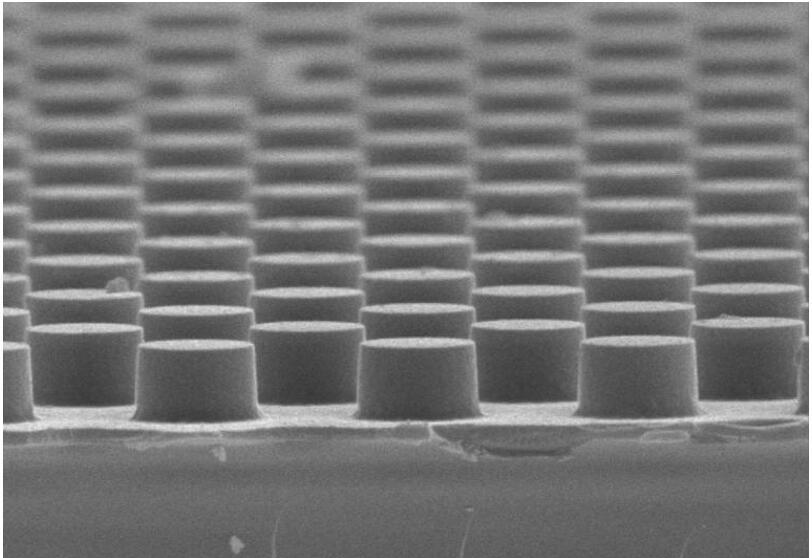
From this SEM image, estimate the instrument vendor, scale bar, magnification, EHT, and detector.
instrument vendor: other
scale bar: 3000 nm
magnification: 30 K X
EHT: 10 kV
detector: SE